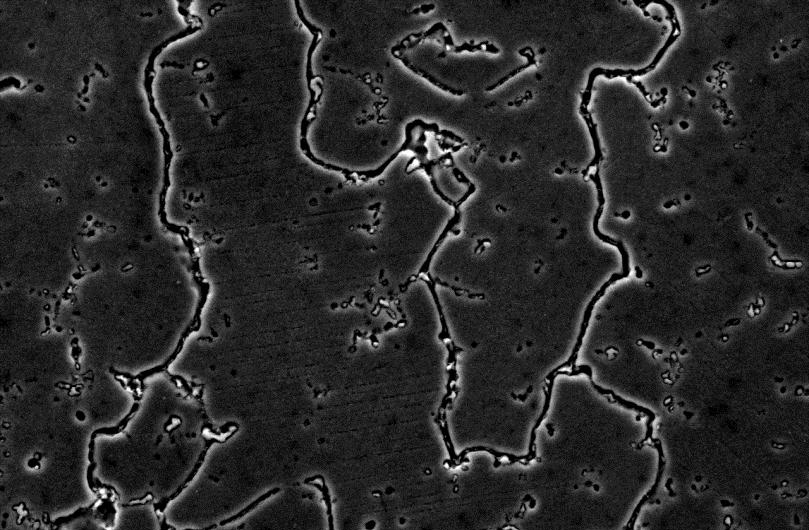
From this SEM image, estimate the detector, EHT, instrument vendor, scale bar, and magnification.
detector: SEI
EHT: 20 kV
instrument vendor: JEOL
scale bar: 10000 nm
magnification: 1.5 K X